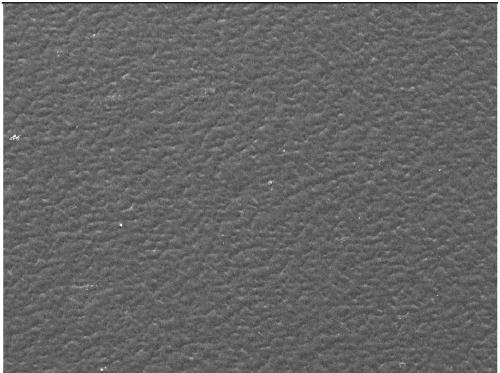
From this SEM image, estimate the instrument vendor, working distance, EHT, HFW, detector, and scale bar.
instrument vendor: Tescan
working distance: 8.51 mm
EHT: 30 kV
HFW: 1020 µm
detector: SE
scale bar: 200000 nm